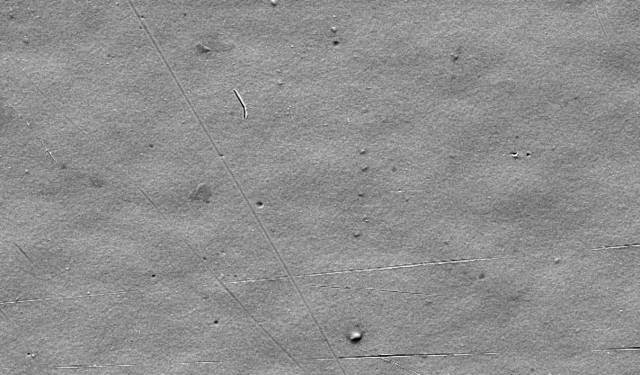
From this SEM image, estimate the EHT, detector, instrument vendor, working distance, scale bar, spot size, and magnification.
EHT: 10 kV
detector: SE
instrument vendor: FEI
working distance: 10.1 mm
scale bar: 10000 nm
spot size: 3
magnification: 2 K X